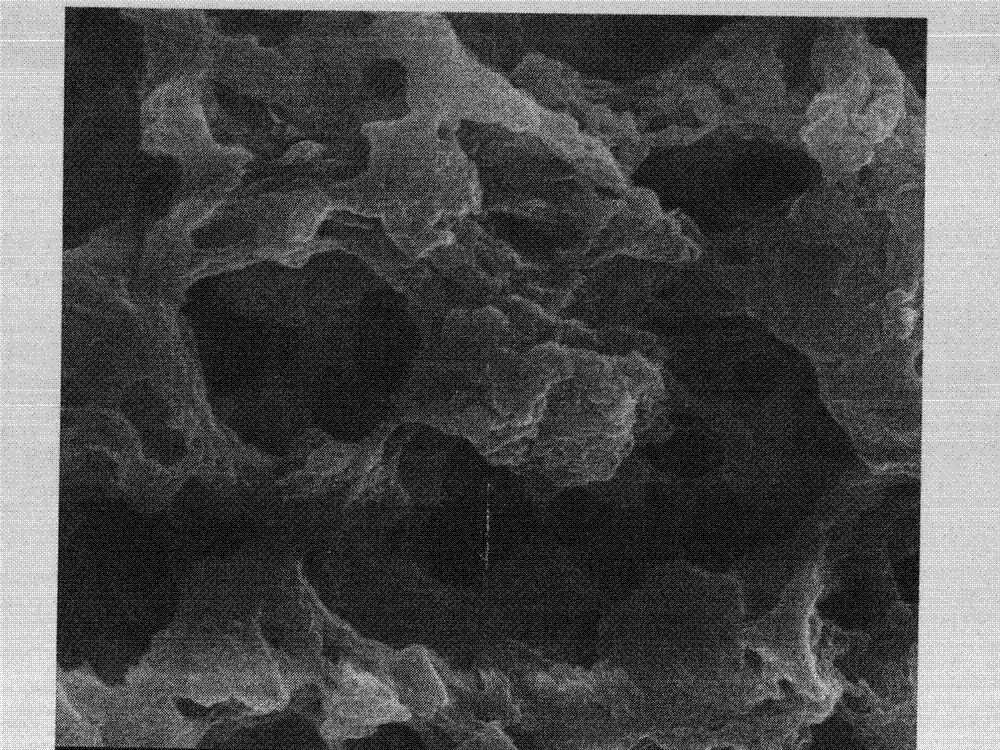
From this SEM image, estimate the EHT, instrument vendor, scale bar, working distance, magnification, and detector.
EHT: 10 kV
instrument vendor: FEI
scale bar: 1000 nm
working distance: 5.7 mm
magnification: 50 K X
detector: TLD